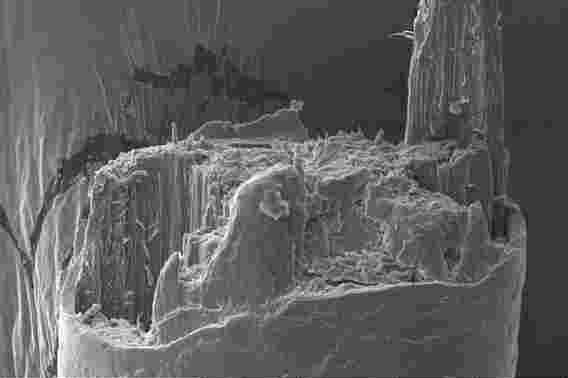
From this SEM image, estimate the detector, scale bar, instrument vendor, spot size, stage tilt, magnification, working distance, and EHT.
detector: ETD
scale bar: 20000 nm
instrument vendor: FEI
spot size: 3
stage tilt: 0°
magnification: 4 K X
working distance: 10.1 mm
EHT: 5 kV